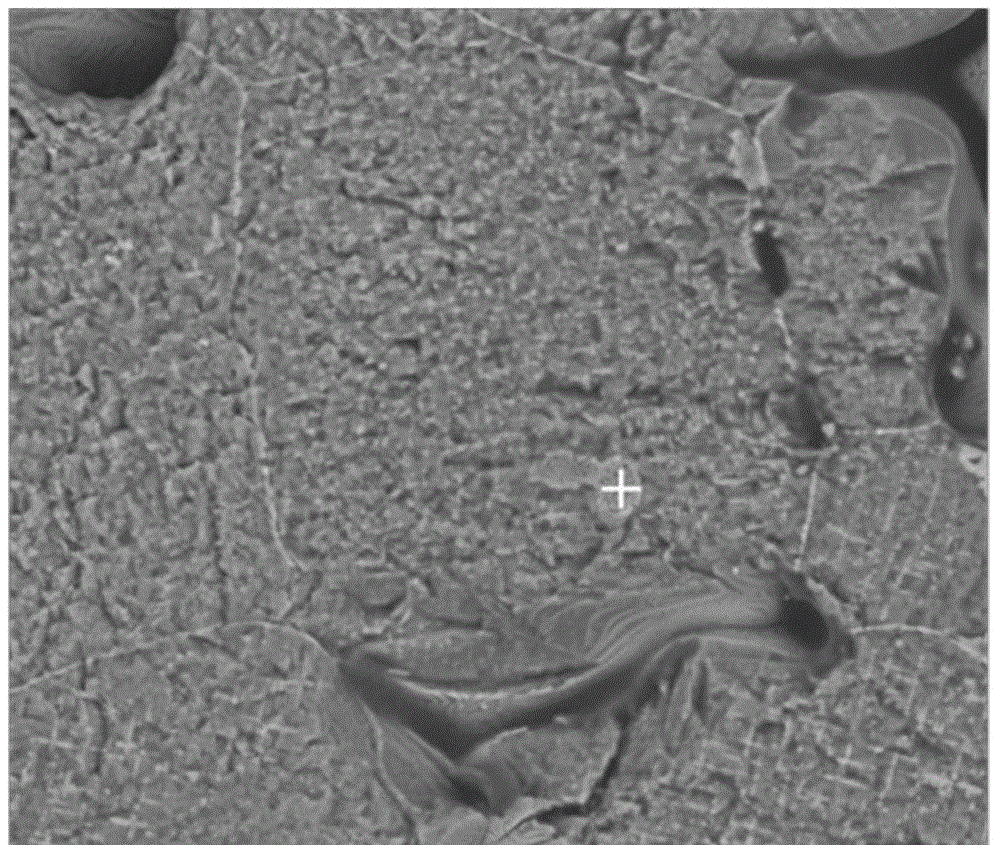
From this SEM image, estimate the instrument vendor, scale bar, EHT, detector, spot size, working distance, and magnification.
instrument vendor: FEI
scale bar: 20000 nm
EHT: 20 kV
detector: BSED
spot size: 3.5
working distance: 6.4 mm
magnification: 2 K X